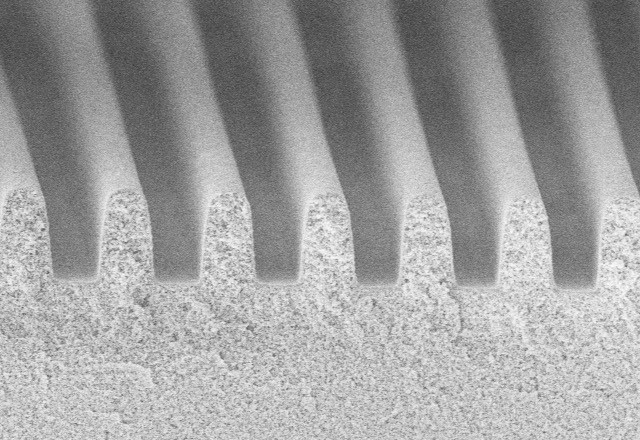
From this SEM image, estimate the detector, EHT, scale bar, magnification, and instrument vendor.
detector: SE(U)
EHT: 5 kV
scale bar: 50000 nm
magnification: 1 K X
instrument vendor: Hitachi